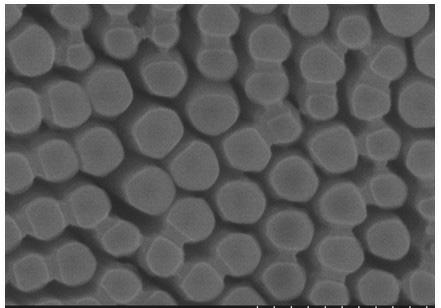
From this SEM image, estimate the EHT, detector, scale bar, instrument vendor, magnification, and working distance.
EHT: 10 kV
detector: SE(M)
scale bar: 500 nm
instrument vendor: Hitachi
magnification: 100 K X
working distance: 8.1 mm